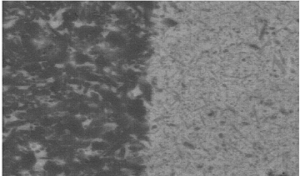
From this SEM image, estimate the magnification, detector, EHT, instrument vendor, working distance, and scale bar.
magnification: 5 K X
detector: BSE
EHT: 20 kV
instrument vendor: FEI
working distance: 4 mm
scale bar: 5000 nm